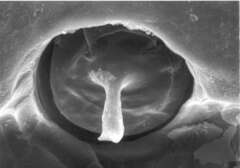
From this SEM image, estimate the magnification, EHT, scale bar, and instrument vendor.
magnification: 1.4 K X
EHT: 20 kV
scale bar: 10000 nm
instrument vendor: JEOL